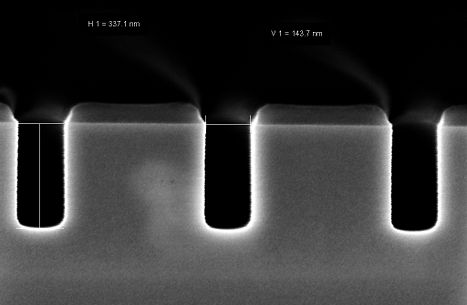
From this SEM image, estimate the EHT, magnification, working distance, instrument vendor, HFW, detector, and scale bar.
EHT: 2 kV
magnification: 200 K X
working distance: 3.3 mm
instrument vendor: Zeiss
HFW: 1.501 µm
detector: InLens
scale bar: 100 nm